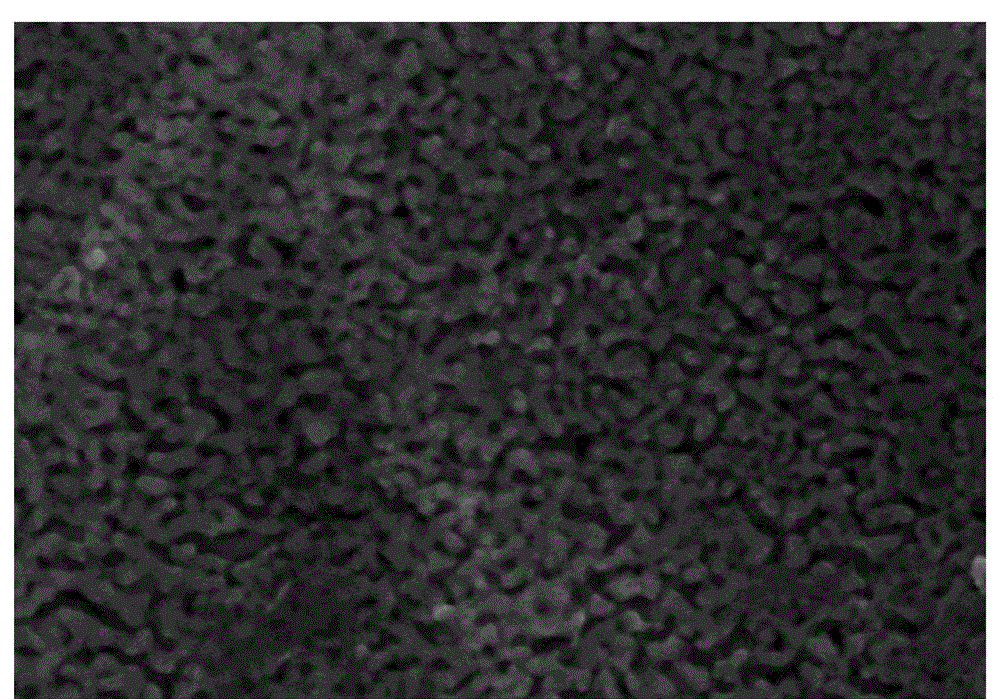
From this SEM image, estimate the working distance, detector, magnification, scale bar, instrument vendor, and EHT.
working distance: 9.8 mm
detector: SE(M)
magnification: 100 K X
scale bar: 500 nm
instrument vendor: Hitachi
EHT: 10 kV